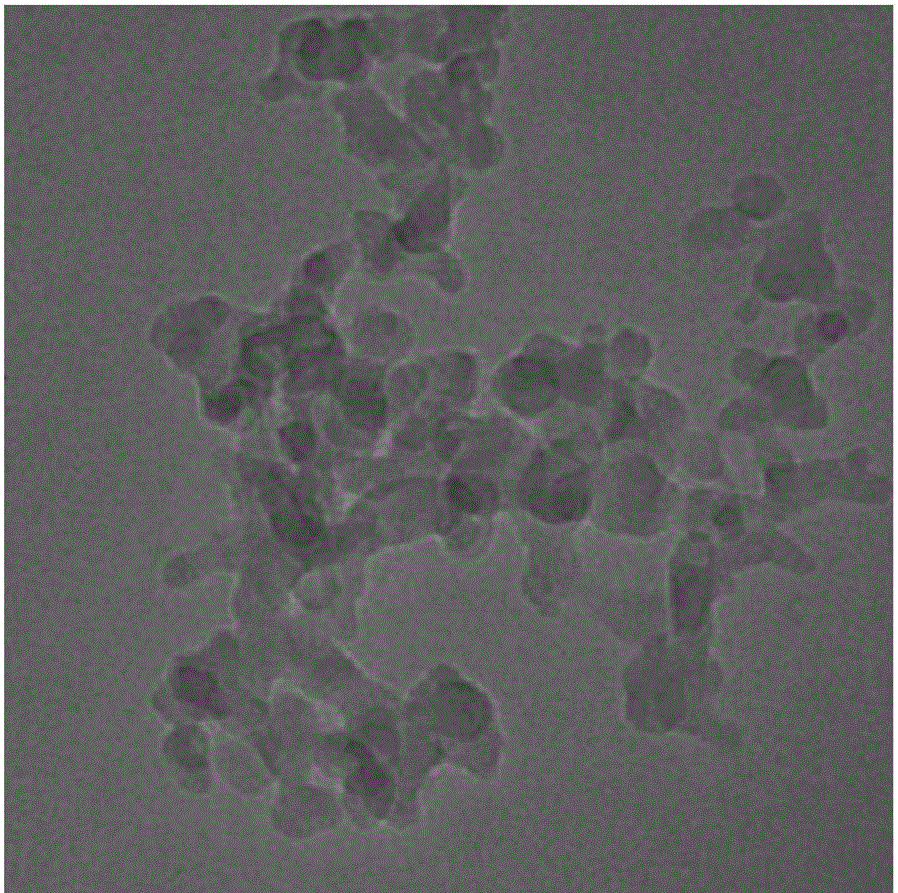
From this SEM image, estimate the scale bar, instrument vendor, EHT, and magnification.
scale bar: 50 nm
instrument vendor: JEOL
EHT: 200 kV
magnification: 250 K X